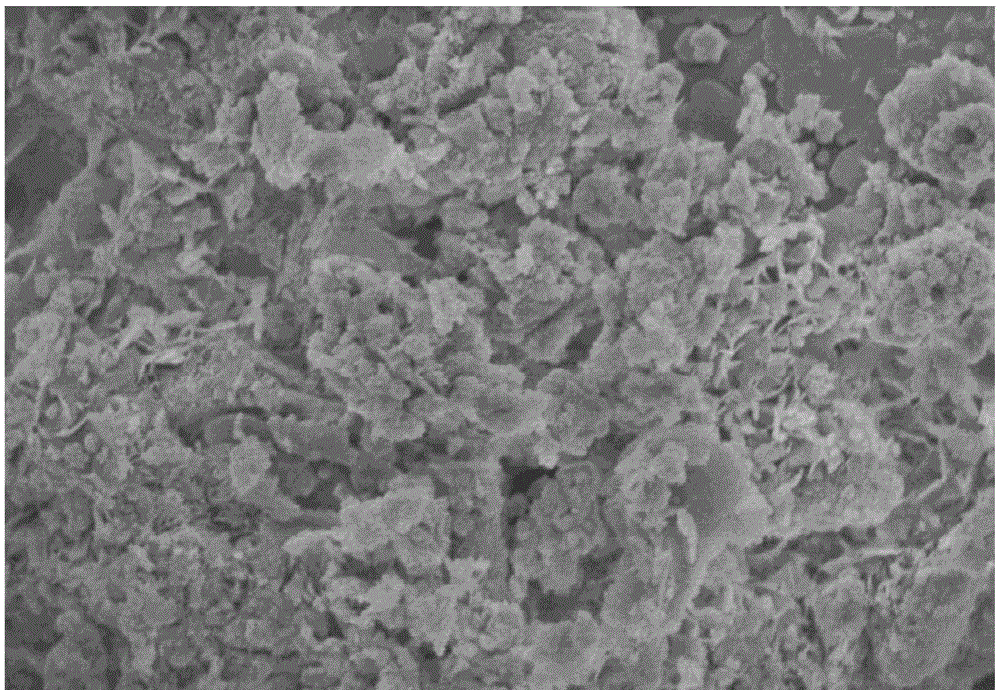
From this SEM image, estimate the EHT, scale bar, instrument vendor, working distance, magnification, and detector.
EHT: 3 kV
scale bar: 2000 nm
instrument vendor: Hitachi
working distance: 10.3 mm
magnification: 20 K X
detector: SE(UL)